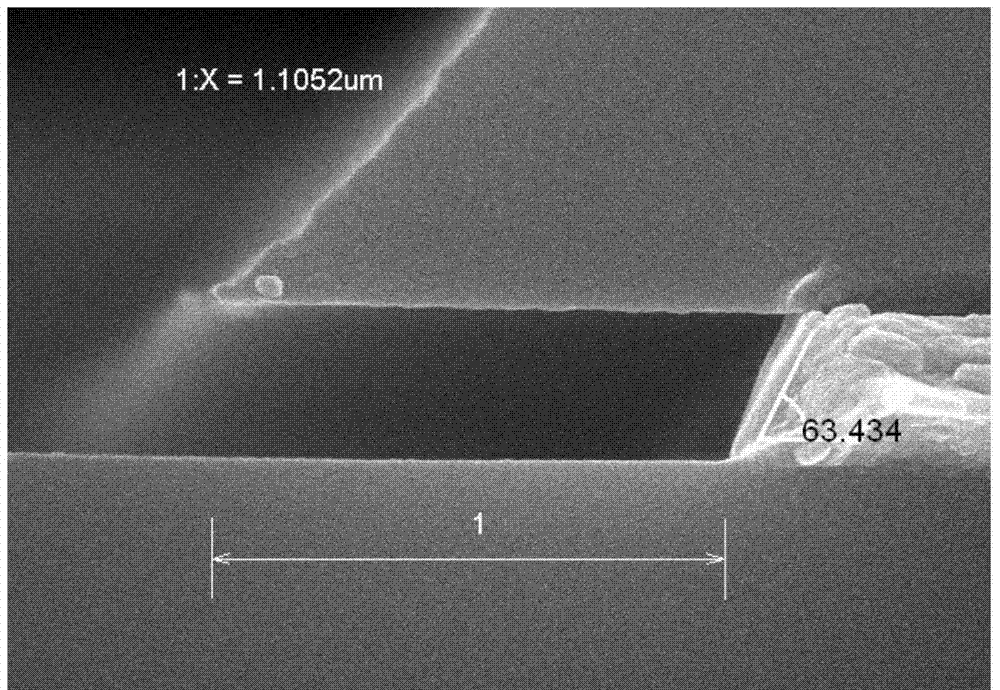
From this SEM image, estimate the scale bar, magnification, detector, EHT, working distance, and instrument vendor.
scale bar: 500 nm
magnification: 60 K X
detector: SE(M)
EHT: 15 kV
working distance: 10.2 mm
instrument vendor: Hitachi